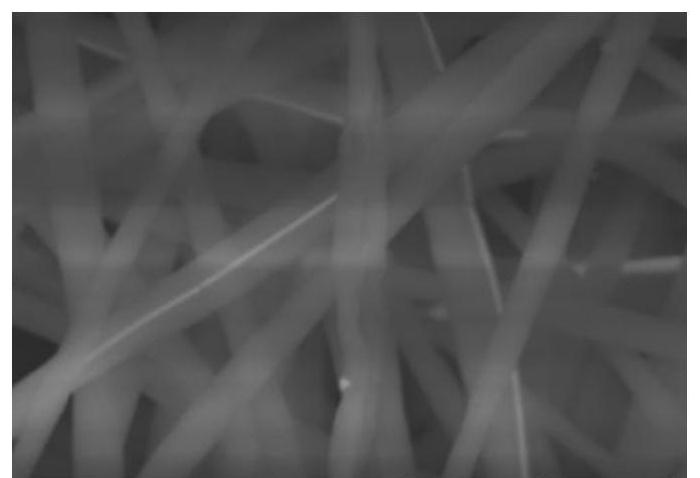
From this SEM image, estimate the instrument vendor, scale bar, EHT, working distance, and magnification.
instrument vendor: Hitachi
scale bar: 5000 nm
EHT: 15 kV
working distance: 16.9 mm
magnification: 10 K X